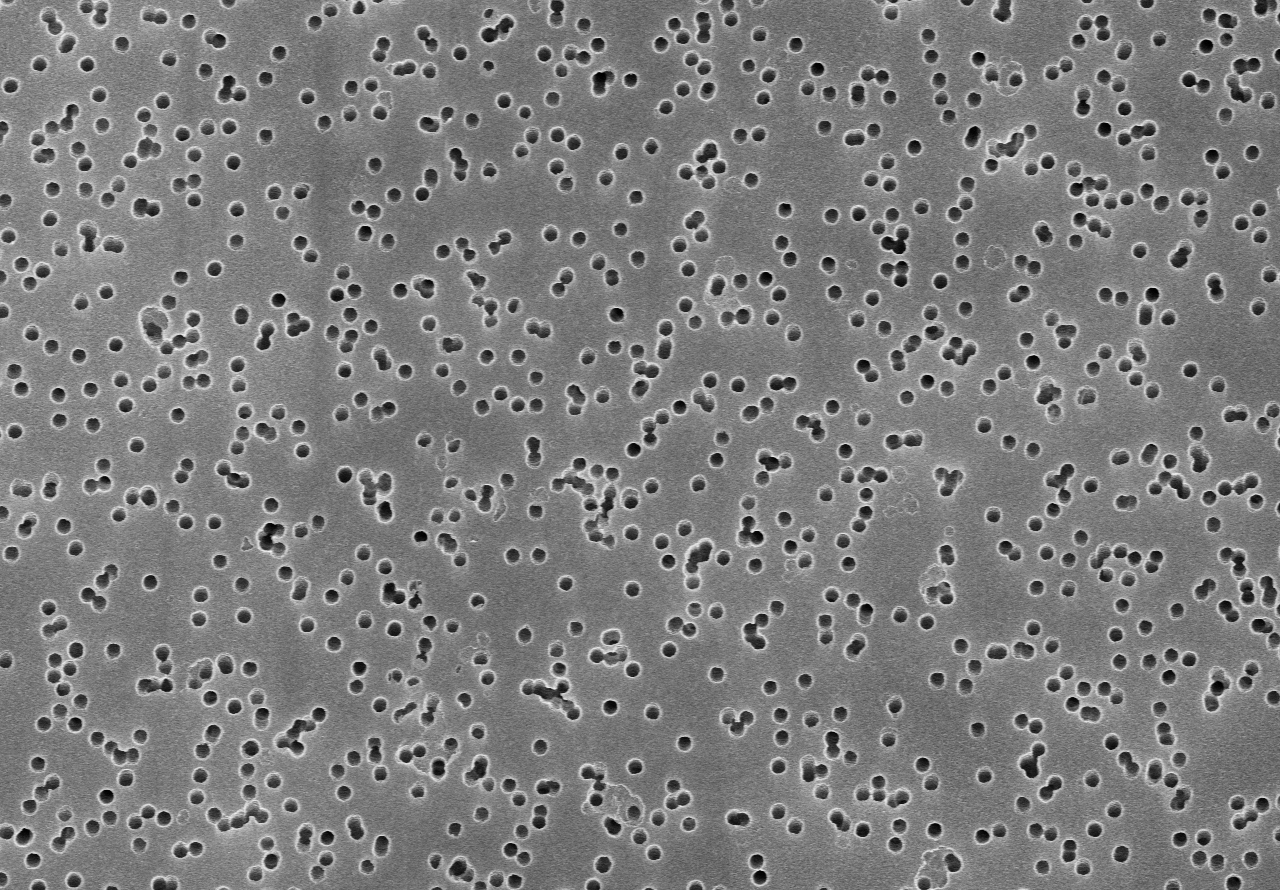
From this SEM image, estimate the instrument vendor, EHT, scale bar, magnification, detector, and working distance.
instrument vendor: Hitachi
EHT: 20 kV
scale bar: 5000 nm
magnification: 6 K X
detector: SE(U)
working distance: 8.8 mm